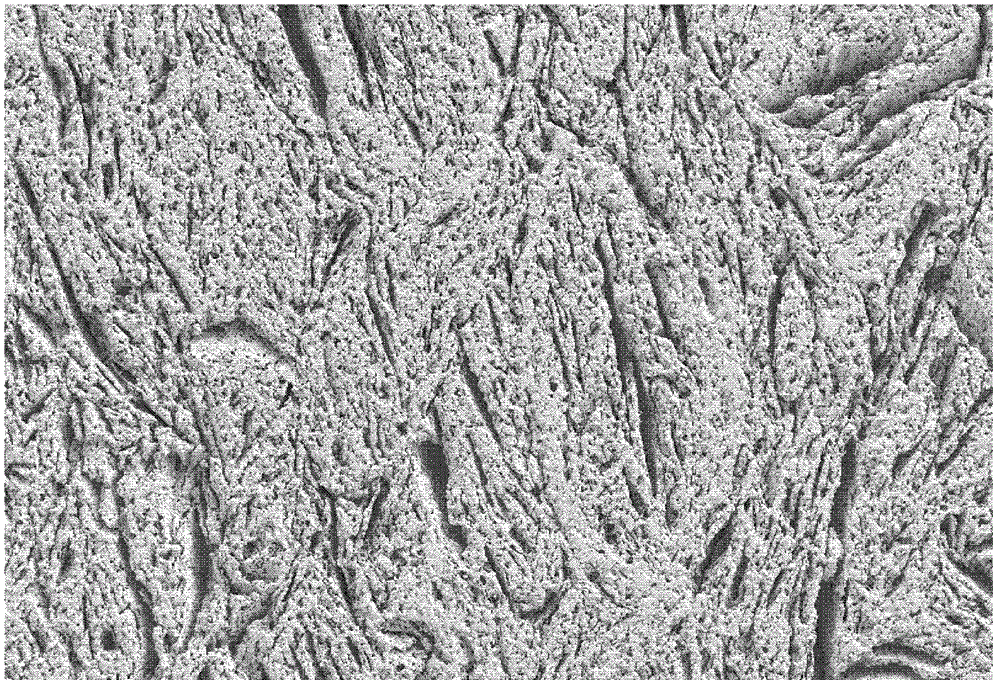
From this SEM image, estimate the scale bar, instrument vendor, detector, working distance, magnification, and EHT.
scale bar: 20000 nm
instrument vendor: Zeiss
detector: SE2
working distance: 5 mm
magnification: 1 K X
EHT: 5 kV